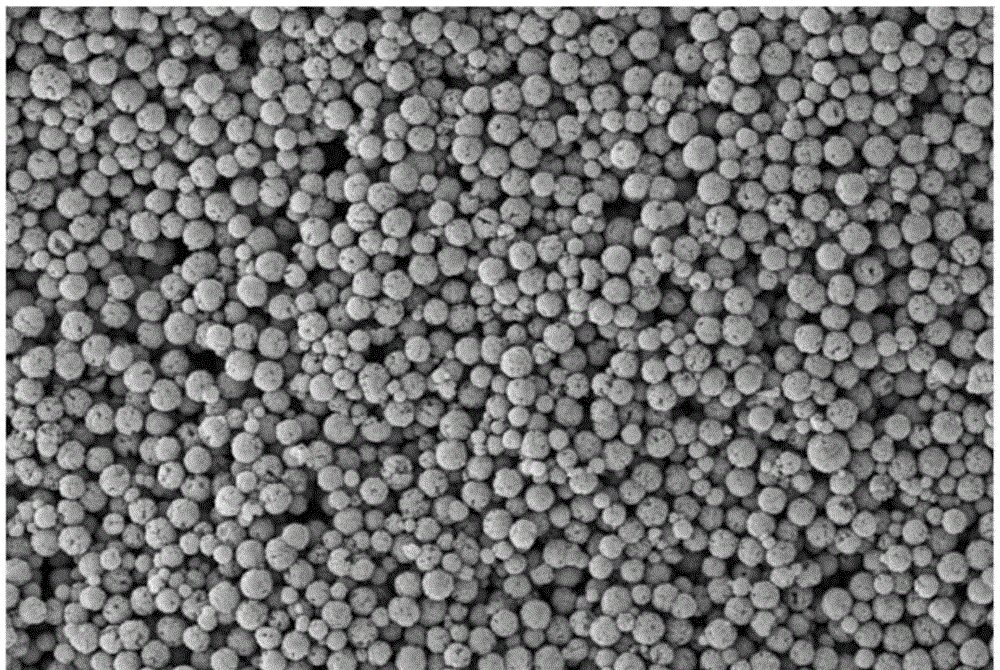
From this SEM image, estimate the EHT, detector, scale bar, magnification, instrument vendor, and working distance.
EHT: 5 kV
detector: SE2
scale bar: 1000 nm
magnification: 10 K X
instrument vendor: Zeiss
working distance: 11.5 mm